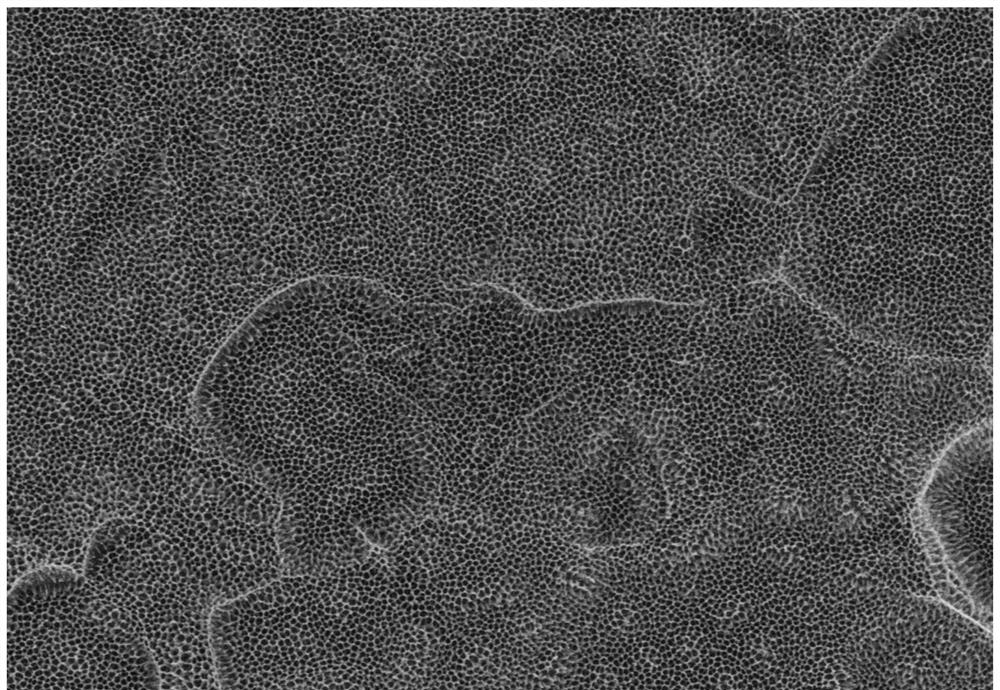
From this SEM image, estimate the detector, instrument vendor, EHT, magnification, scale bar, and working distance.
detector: SE(U)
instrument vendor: Hitachi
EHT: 5 kV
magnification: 20 K X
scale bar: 2000 nm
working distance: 5.2 mm